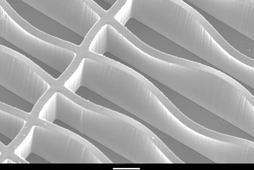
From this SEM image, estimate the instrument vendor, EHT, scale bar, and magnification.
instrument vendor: JEOL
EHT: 10 kV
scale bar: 50000 nm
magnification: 0.27 K X